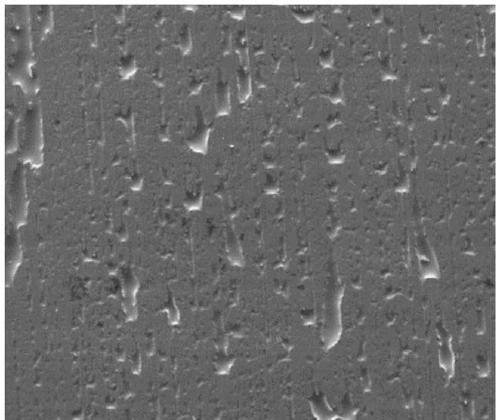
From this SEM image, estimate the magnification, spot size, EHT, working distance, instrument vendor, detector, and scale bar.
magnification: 0.5 K X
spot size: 5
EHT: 20 kV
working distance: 14.6 mm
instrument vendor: FEI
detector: ETD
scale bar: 200000 nm